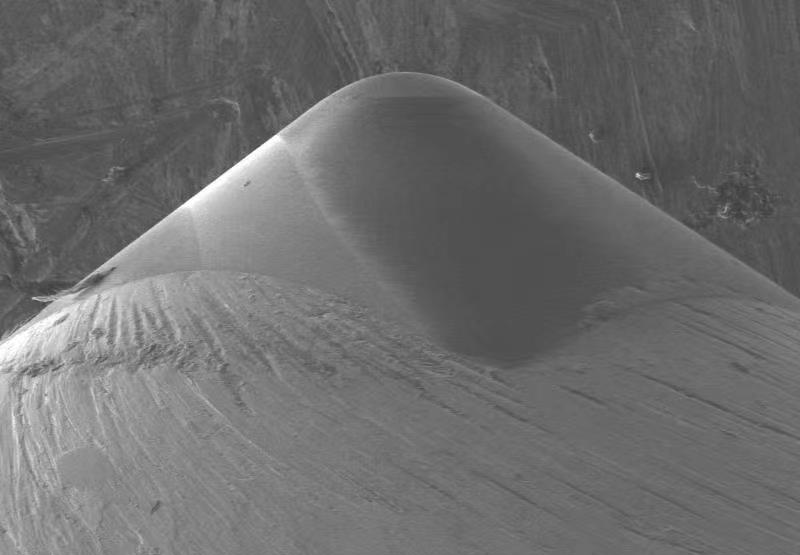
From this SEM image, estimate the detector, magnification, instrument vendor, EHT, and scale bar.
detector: LM(UL)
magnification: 0.1 K X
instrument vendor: Hitachi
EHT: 10 kV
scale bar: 500000 nm